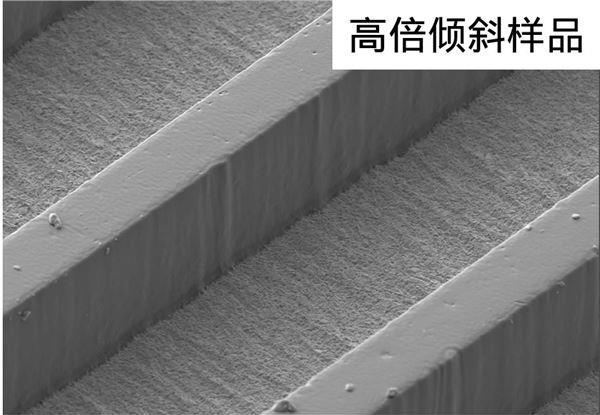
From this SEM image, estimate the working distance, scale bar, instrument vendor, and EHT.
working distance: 7.8 mm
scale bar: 50000 nm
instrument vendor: Zeiss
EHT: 5 kV